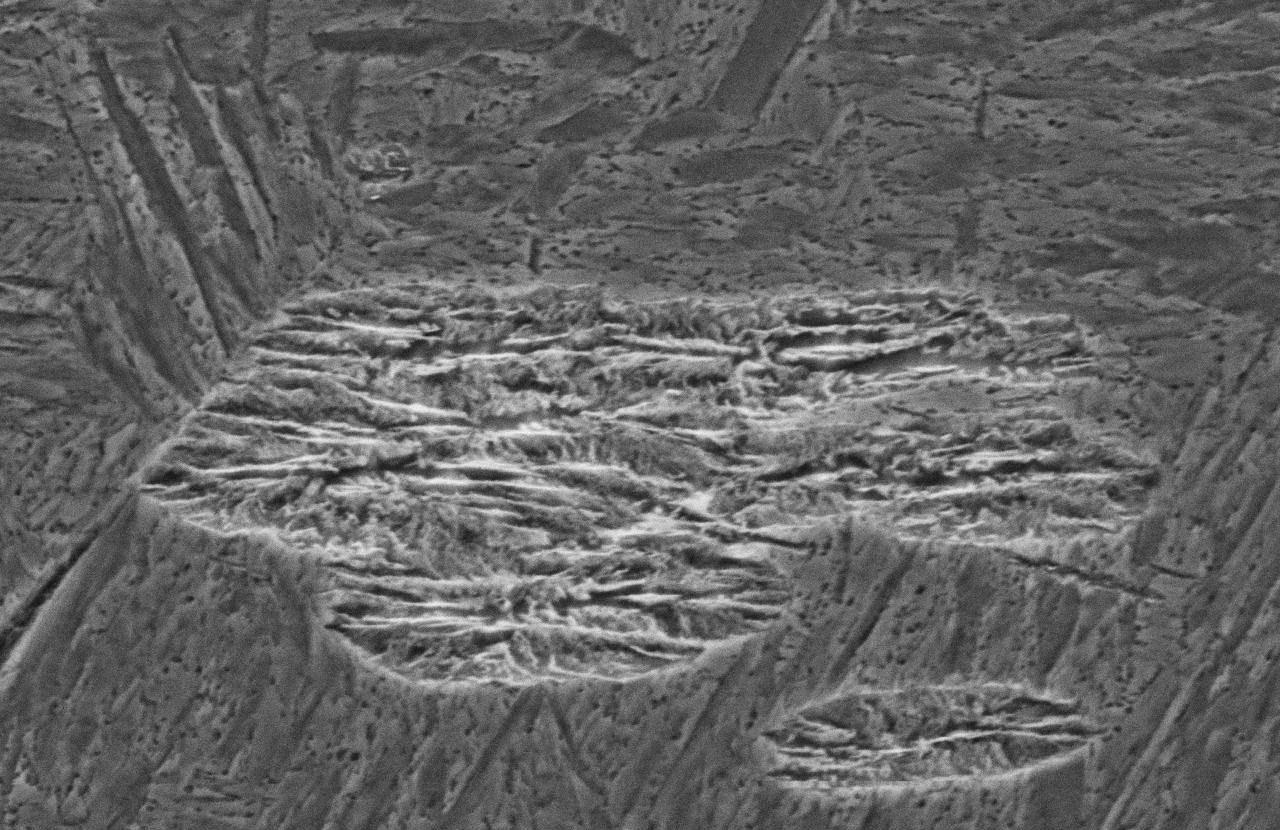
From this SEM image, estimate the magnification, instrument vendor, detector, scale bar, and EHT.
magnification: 4 K X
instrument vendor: JEOL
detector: SEI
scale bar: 5000 nm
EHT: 15 kV